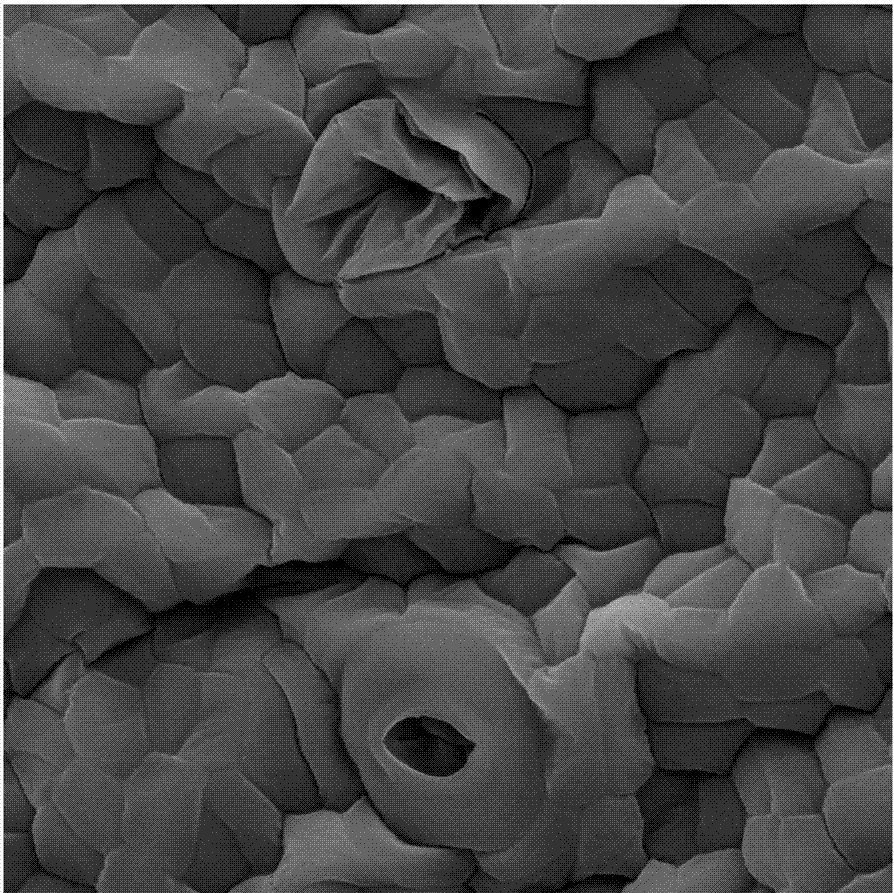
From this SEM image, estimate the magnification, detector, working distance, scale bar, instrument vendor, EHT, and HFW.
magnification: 2 K X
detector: SE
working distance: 17.59 mm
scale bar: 20000 nm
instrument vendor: Tescan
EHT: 30 kV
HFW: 108 µm